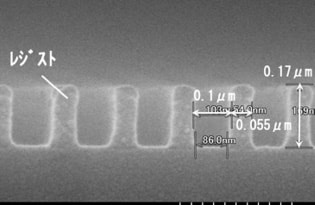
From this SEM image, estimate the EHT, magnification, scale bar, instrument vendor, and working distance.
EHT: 5 kV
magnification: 150 K X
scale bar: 300 nm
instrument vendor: Hitachi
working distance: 2.6 mm